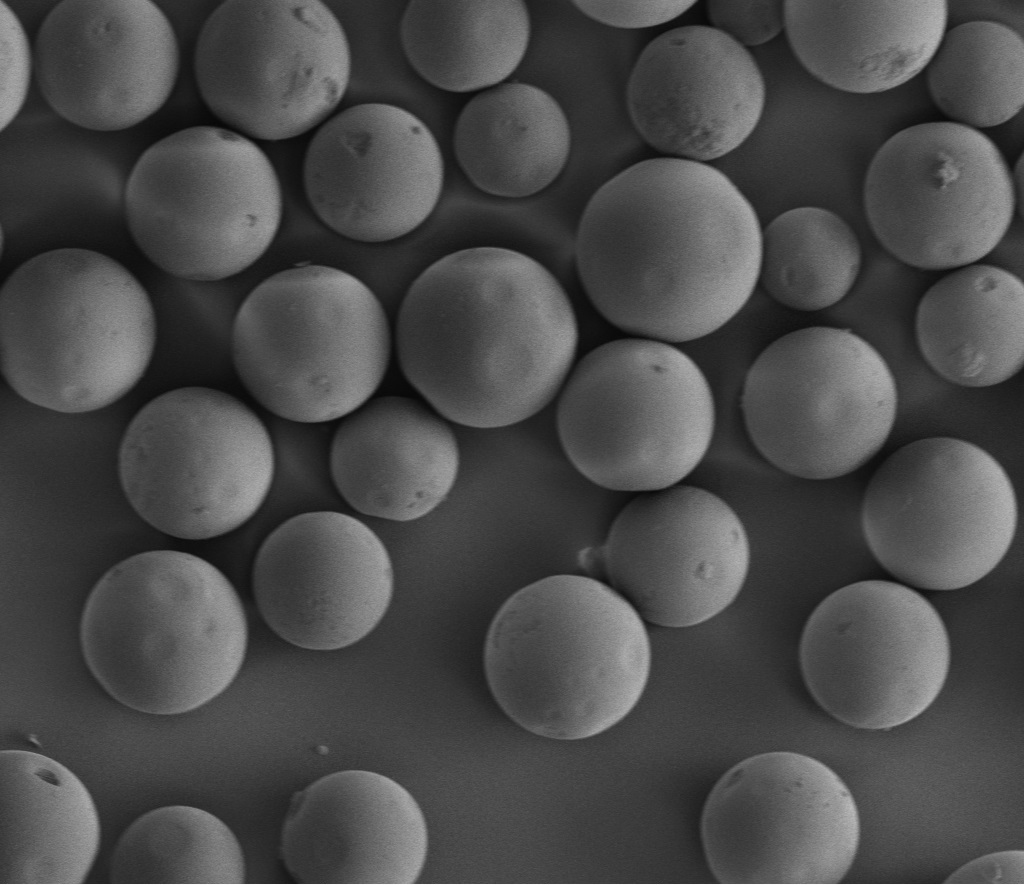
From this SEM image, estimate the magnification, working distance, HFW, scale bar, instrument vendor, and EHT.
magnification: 0.28 K X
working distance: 4.9 mm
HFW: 533 µm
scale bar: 100000 nm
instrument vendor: FEI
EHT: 3 kV